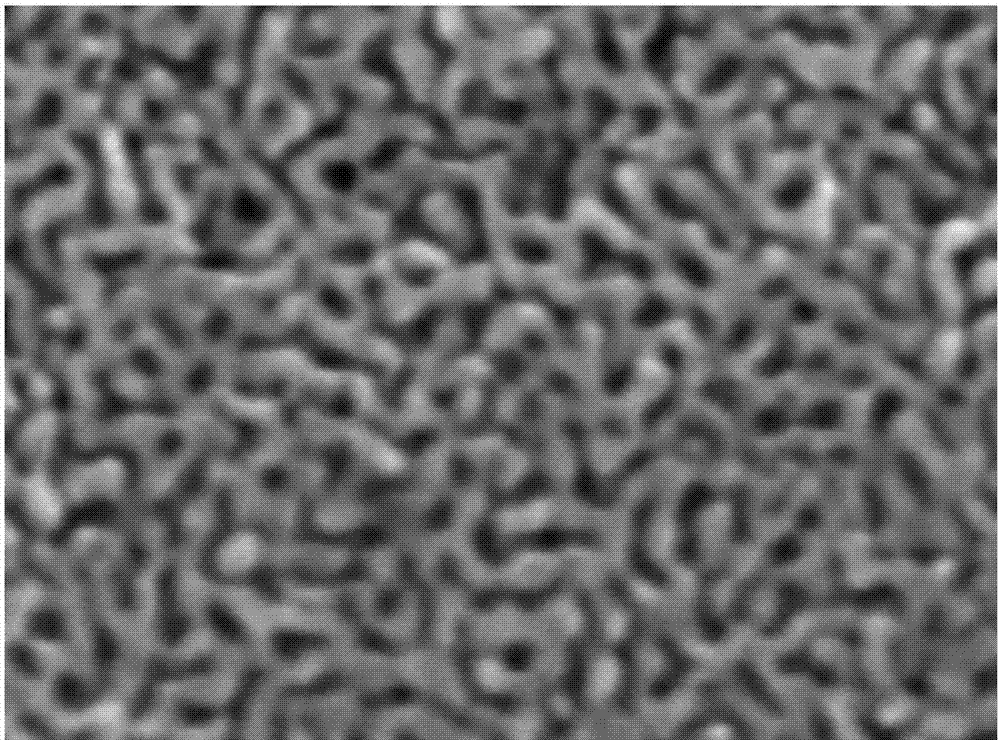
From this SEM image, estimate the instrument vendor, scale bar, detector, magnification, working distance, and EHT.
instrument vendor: JEOL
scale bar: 10 nm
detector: UED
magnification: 300 K X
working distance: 4 mm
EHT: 10 kV